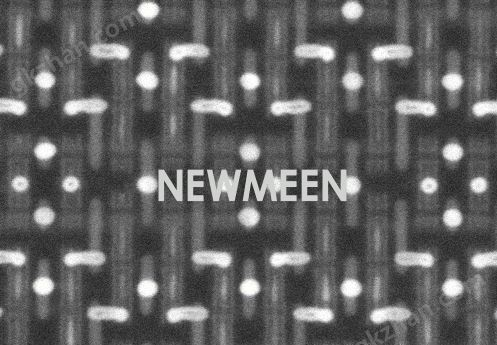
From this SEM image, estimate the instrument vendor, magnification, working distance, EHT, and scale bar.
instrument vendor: Hitachi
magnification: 250 K X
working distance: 7.1 mm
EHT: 30 kV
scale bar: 200 nm